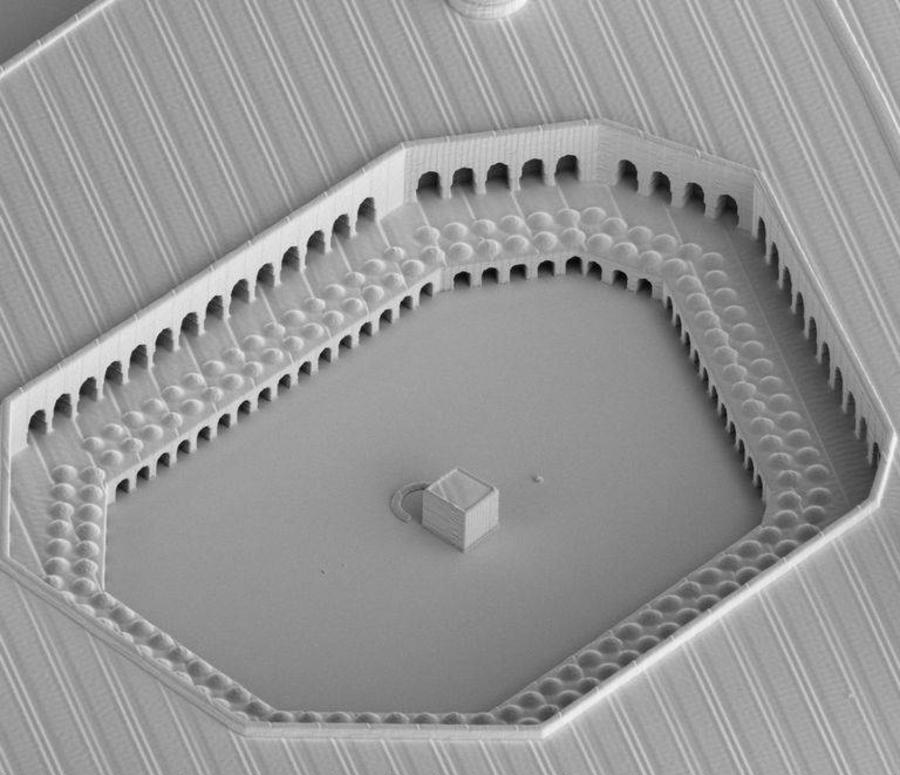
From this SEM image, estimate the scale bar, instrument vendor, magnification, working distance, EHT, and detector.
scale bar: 50000 nm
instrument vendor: FEI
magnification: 0.35 K X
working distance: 4.3 mm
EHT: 2 kV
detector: ETD